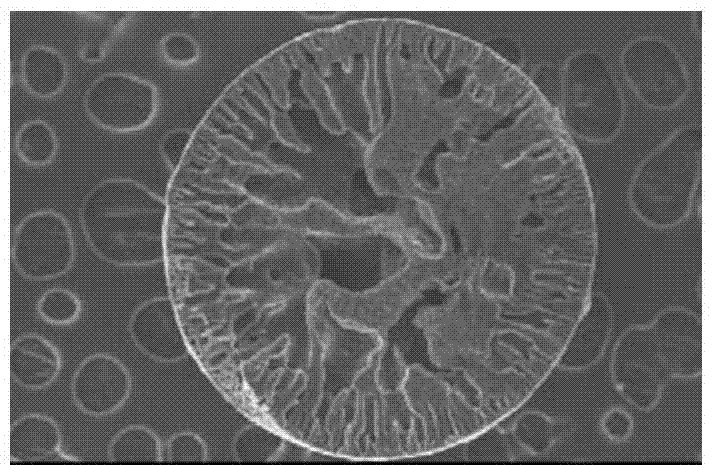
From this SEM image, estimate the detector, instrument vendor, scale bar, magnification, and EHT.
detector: SEI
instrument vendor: JEOL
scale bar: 500000 nm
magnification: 0.05 K X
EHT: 30 kV